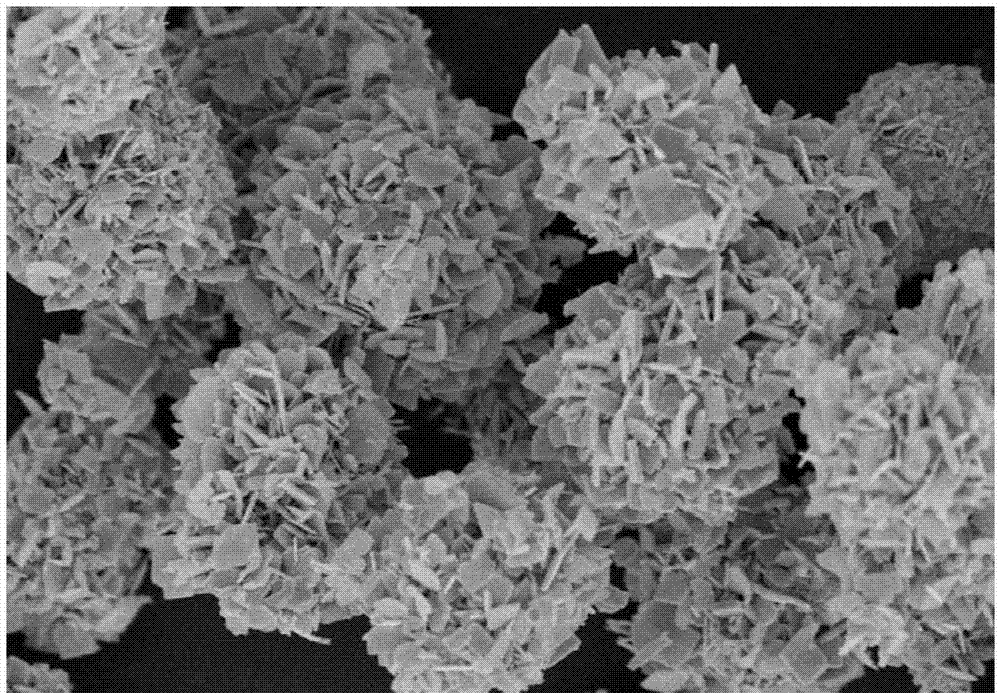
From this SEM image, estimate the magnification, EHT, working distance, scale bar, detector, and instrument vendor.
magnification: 8 K X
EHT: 5 kV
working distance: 7.7 mm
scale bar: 5000 nm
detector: SE(M)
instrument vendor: Hitachi